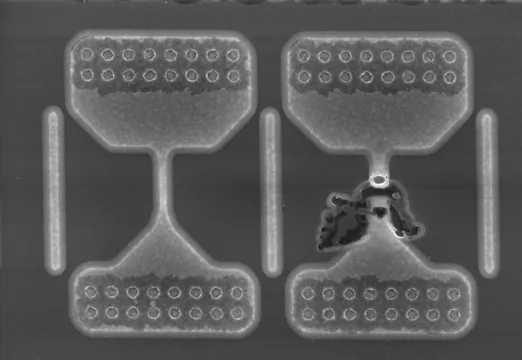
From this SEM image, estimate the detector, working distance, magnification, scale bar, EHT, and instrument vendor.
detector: SE(M)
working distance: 9.1 mm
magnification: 15 K X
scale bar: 3000 nm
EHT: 10 kV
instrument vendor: Hitachi